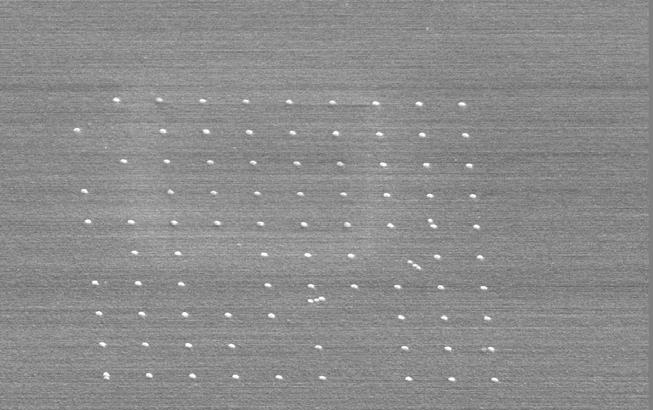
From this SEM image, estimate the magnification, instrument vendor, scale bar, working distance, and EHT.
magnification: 17 K X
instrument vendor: JEOL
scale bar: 1000 nm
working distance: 14 mm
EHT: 15 kV